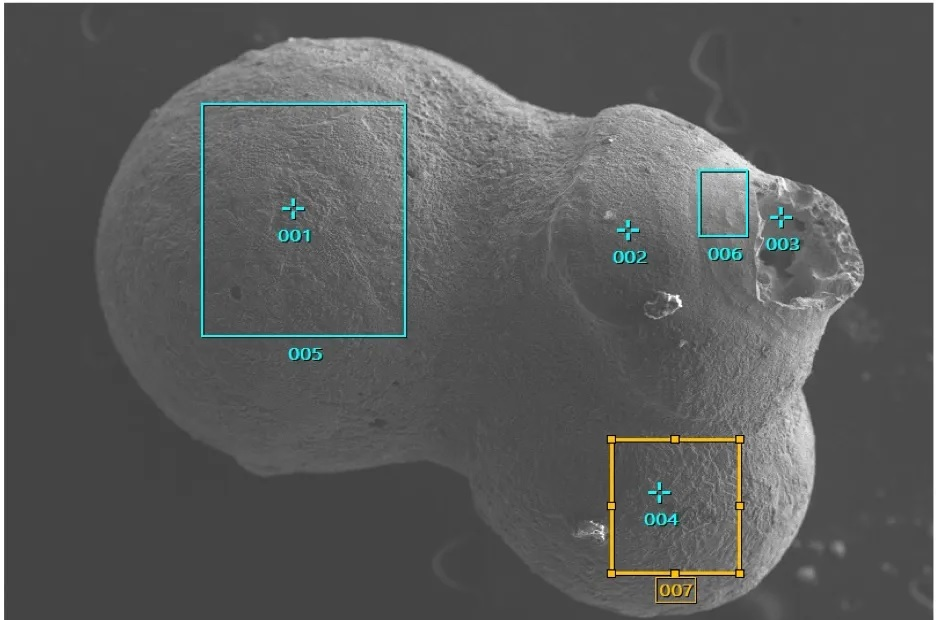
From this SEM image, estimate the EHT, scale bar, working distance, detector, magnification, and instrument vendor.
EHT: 15 kV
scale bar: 100000 nm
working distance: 11.1 mm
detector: SEI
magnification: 0.07 K X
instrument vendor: JEOL